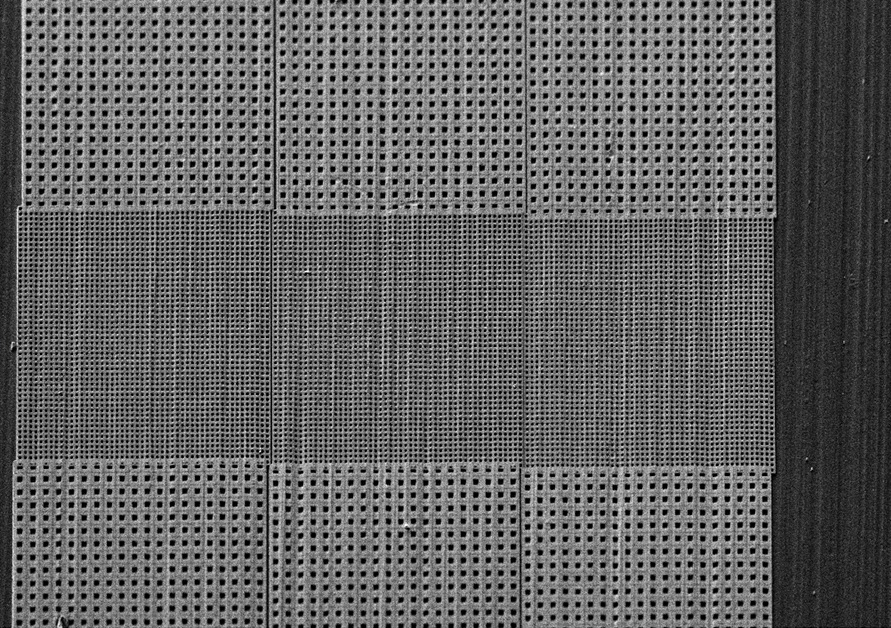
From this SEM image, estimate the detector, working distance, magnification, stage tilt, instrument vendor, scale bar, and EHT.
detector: SE2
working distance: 7.4 mm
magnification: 0.419 K X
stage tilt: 45°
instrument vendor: Zeiss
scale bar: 30000 nm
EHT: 5 kV